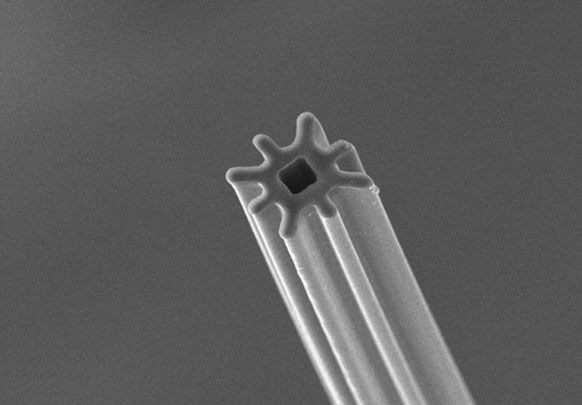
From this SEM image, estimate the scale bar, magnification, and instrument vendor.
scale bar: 10000 nm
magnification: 1 K X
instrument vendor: Hitachi